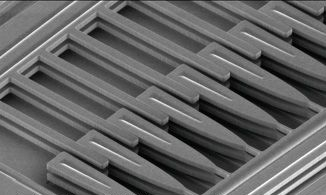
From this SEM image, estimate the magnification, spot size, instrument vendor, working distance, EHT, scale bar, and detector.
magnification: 1.3 K X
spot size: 4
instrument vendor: FEI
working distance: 22.4 mm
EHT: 3 kV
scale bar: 20000 nm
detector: SE